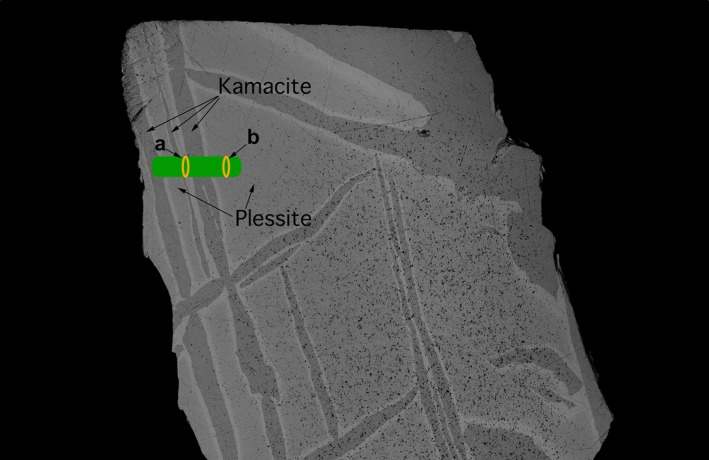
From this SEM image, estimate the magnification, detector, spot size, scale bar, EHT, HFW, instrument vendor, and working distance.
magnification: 0.271 K X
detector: CBS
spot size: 5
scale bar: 400000 nm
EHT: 10 kV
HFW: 1530 µm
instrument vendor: FEI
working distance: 9.6 mm